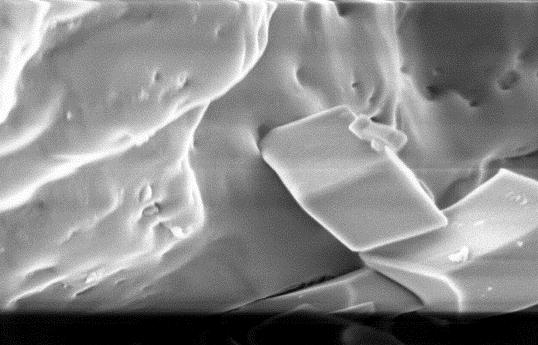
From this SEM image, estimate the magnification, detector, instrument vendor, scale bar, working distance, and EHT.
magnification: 11.64 K X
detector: InLens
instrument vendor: Zeiss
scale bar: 300 nm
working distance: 4 mm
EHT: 5 kV